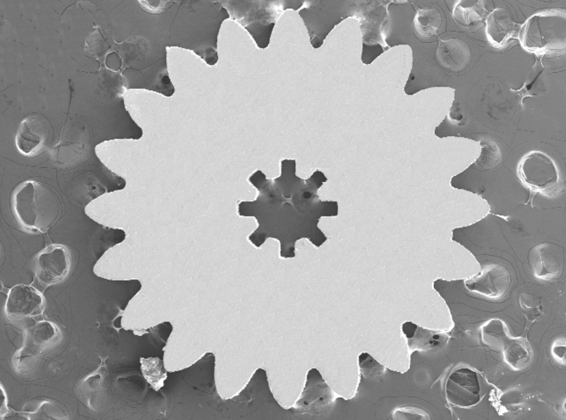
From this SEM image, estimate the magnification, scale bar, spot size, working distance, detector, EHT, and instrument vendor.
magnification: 0.04 K X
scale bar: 500000 nm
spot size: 3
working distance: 5.3 mm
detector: SE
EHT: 20 kV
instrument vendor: FEI